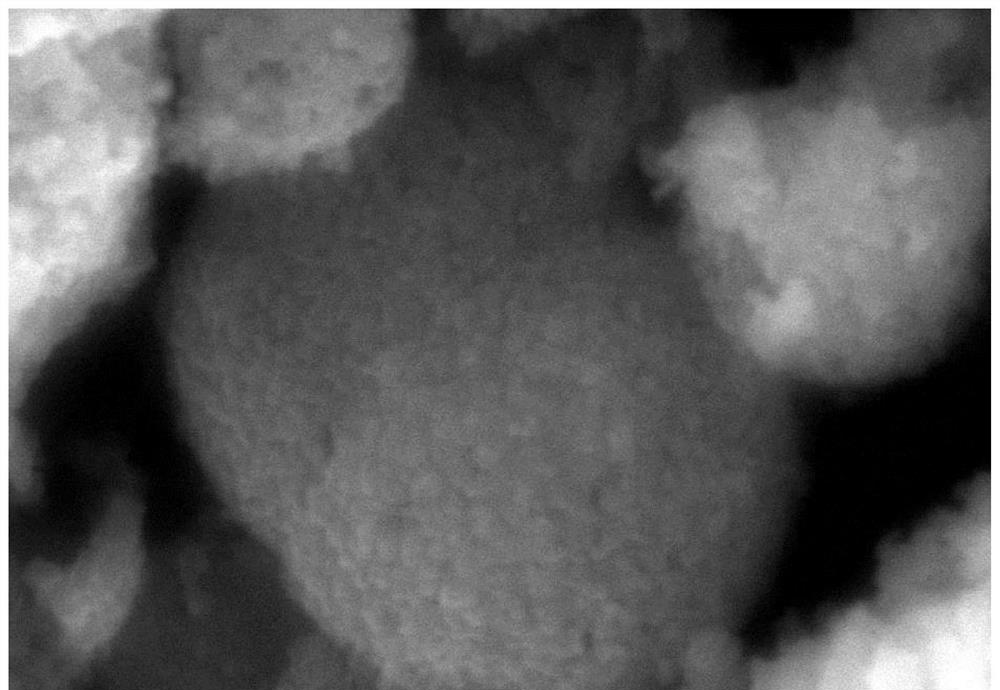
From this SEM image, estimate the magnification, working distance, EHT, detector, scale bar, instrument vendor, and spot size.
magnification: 30 K X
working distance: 10 mm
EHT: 25 kV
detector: SEI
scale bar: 500 nm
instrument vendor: JEOL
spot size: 30